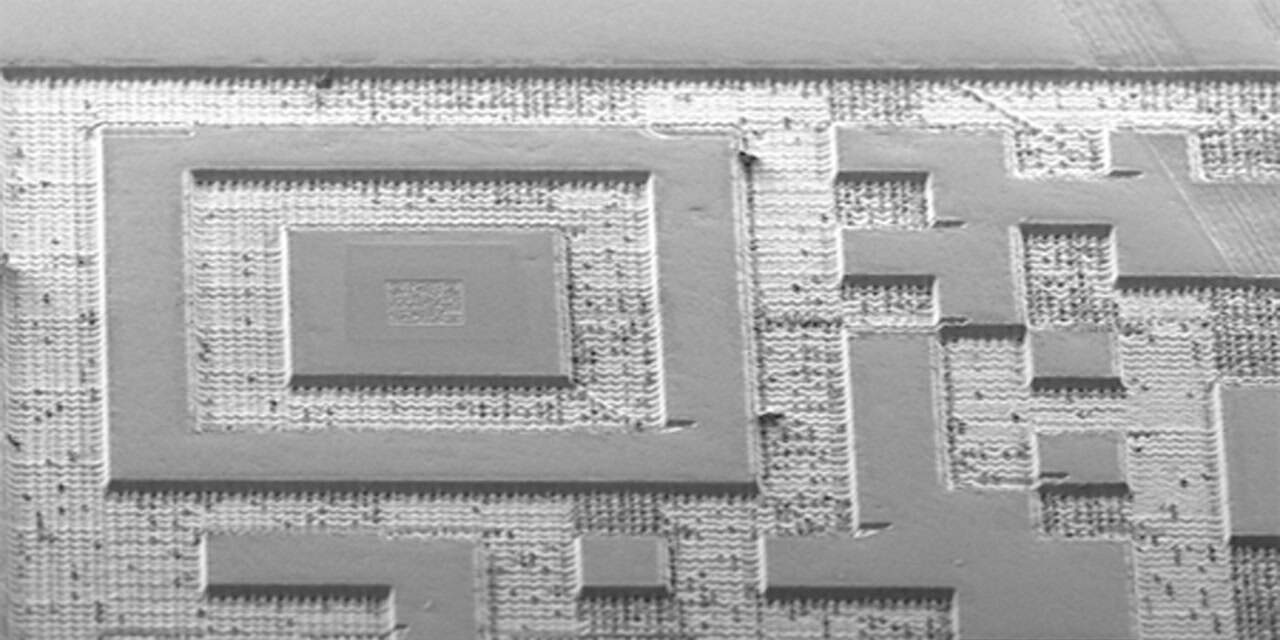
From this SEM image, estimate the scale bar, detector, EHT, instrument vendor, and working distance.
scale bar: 100000 nm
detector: SE2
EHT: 10 kV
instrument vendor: Zeiss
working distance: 4.8 mm